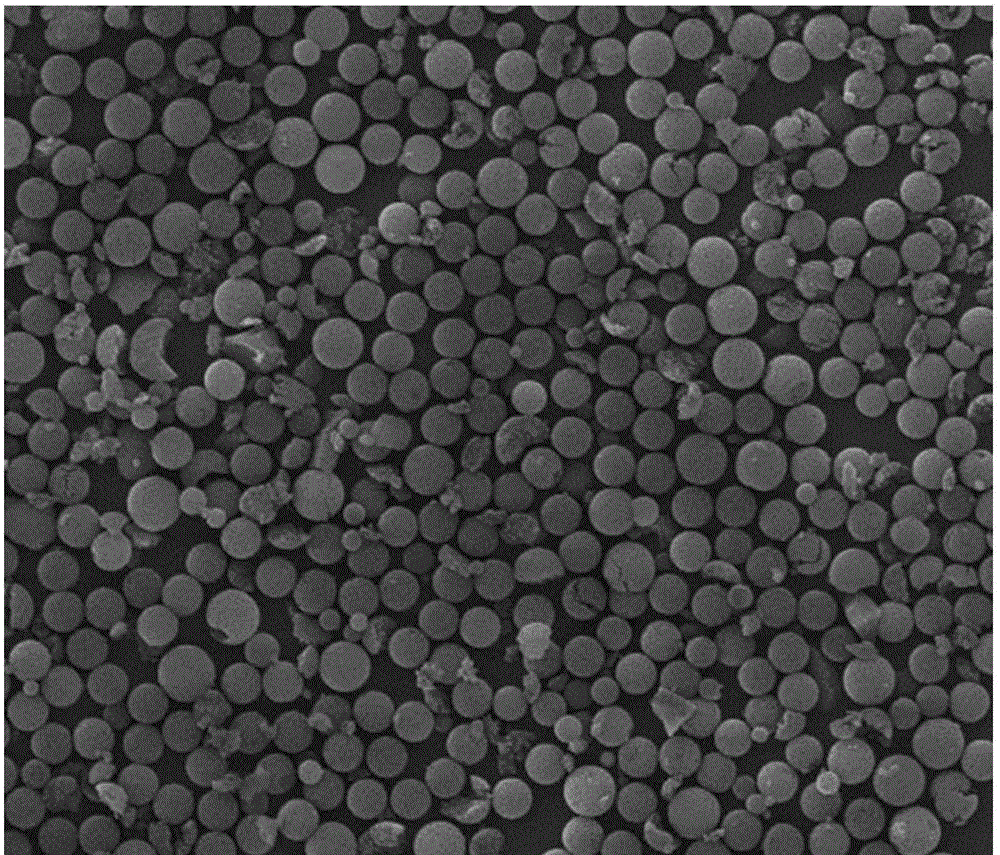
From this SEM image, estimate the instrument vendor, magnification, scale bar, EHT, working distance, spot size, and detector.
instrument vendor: FEI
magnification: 0.6 K X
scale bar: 50000 nm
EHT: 30 kV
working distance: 23.9 mm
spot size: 4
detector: ETD